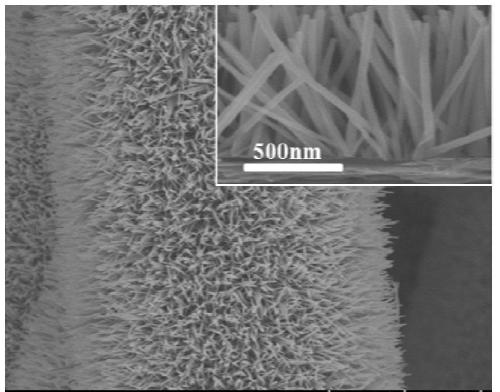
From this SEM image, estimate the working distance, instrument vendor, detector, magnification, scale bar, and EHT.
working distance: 10.6 mm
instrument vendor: Hitachi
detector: SE(UL)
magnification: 7 K X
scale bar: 5000 nm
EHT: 5 kV